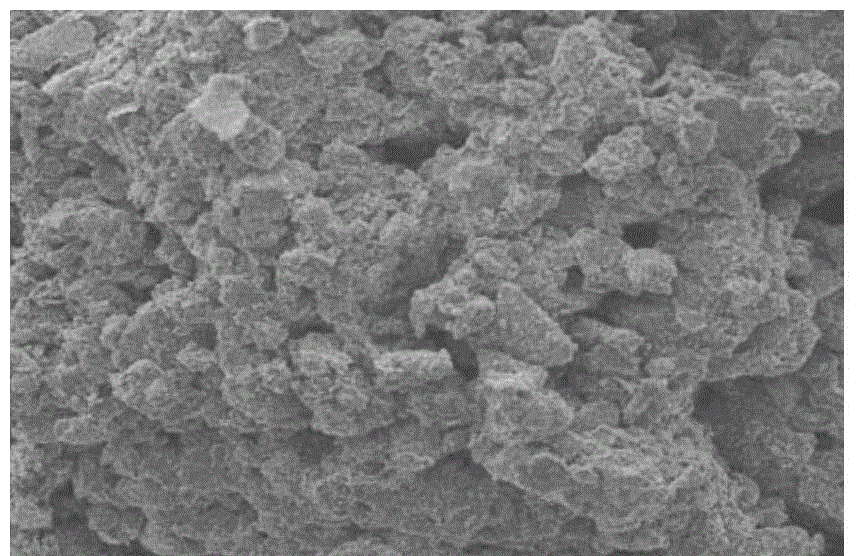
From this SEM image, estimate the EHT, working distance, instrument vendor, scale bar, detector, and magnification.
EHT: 20 kV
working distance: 12.3 mm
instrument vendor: FEI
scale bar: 100000 nm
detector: ETD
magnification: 0.3 K X